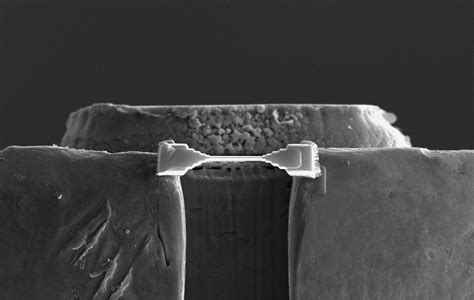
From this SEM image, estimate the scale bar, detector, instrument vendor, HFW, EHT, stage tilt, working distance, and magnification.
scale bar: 5000 nm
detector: ETD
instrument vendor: FEI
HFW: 36.3 µm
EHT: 15 kV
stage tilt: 52°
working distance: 4 mm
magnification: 3.5 K X